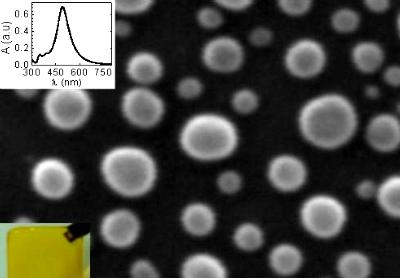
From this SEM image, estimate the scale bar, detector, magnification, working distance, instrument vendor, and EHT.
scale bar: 10 nm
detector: SEI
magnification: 400 K X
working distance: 10 mm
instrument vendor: JEOL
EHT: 30 kV